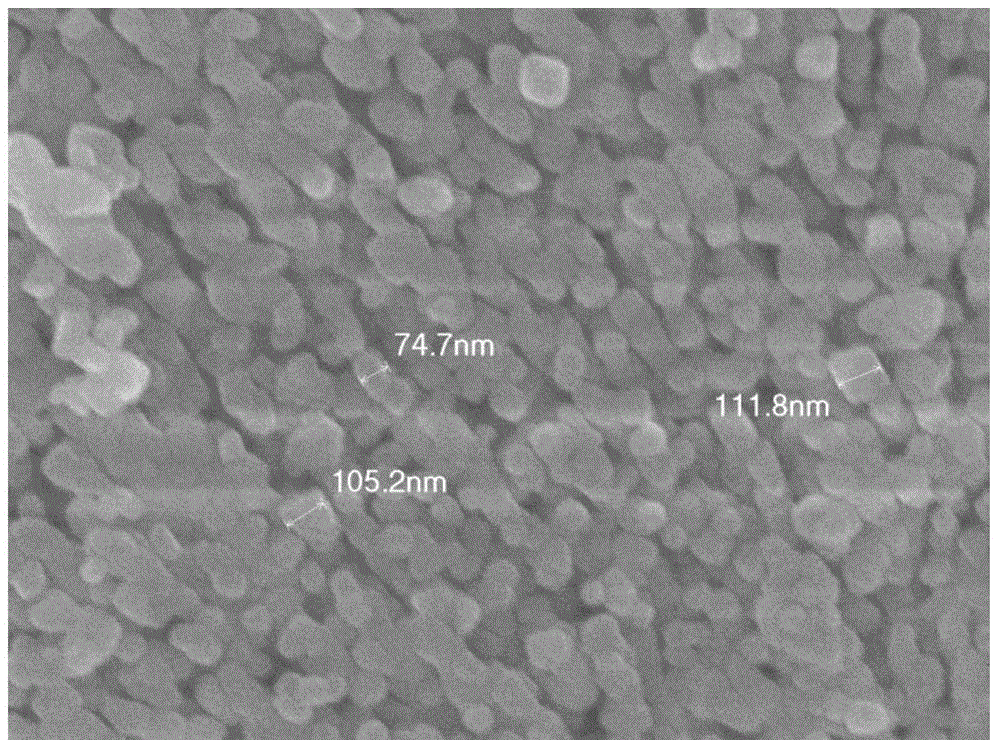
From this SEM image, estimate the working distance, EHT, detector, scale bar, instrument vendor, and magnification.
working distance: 7.7 mm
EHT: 10 kV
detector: SEI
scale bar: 100 nm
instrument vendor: JEOL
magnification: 50 K X